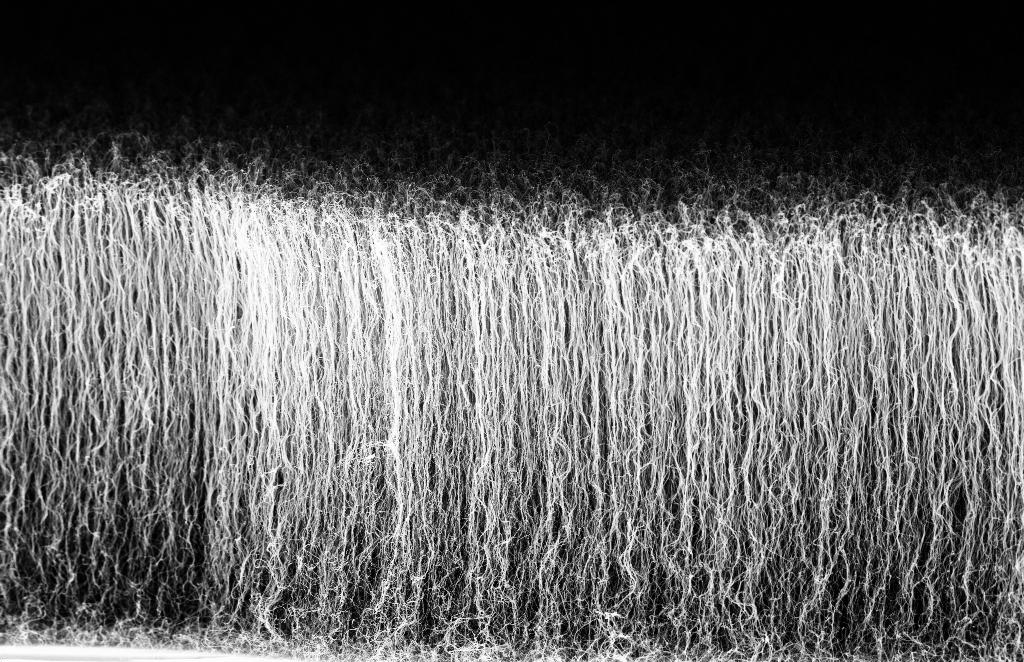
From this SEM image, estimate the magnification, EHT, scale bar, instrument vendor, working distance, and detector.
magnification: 10 K X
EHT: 5 kV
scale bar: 1000 nm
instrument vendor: Zeiss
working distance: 6 mm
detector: InLens